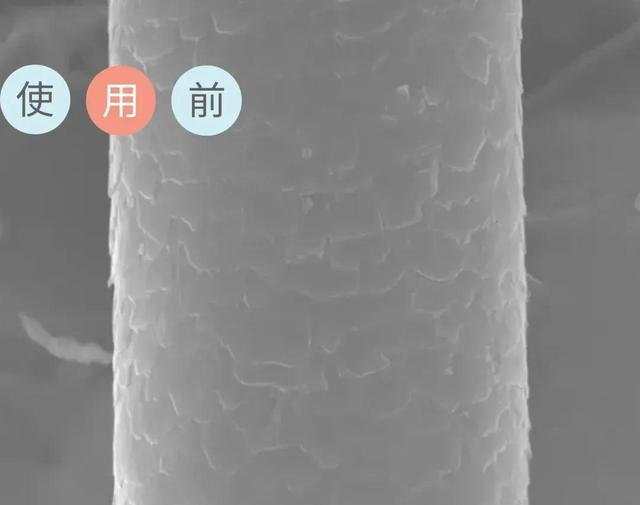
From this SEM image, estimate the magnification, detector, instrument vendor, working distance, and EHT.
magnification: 1 K X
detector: SED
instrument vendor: JEOL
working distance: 9.2 mm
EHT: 20 kV